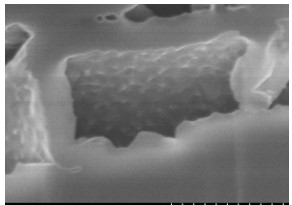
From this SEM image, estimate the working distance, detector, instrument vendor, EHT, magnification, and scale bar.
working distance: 5 mm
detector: SE(M)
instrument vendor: Hitachi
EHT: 3 kV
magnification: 100 K X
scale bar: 500 nm